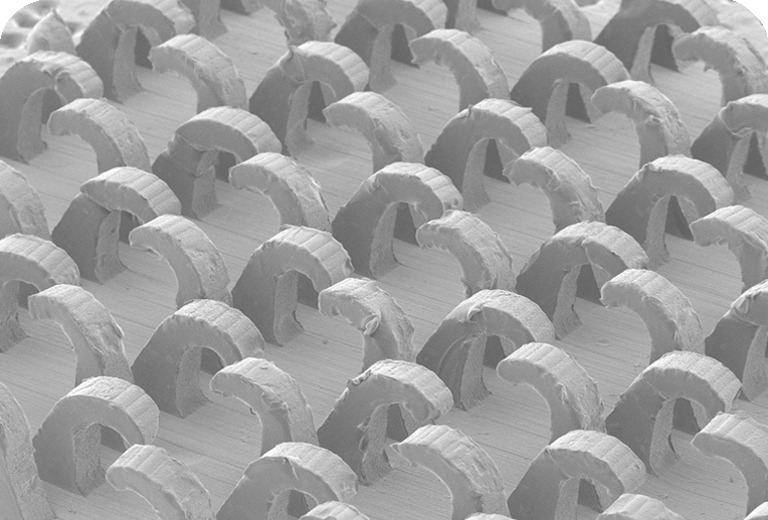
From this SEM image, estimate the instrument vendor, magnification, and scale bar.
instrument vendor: JEOL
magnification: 0.025 K X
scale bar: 1000000 nm